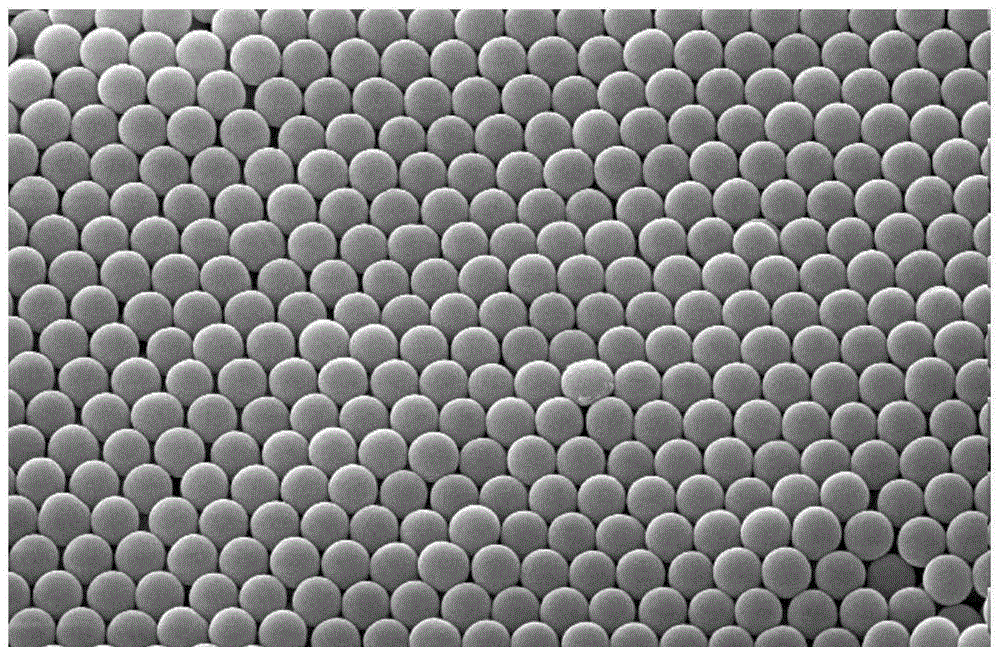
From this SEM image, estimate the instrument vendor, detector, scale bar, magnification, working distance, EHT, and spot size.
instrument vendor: FEI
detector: SE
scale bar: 2000 nm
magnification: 10 K X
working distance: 10 mm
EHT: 15 kV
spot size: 3